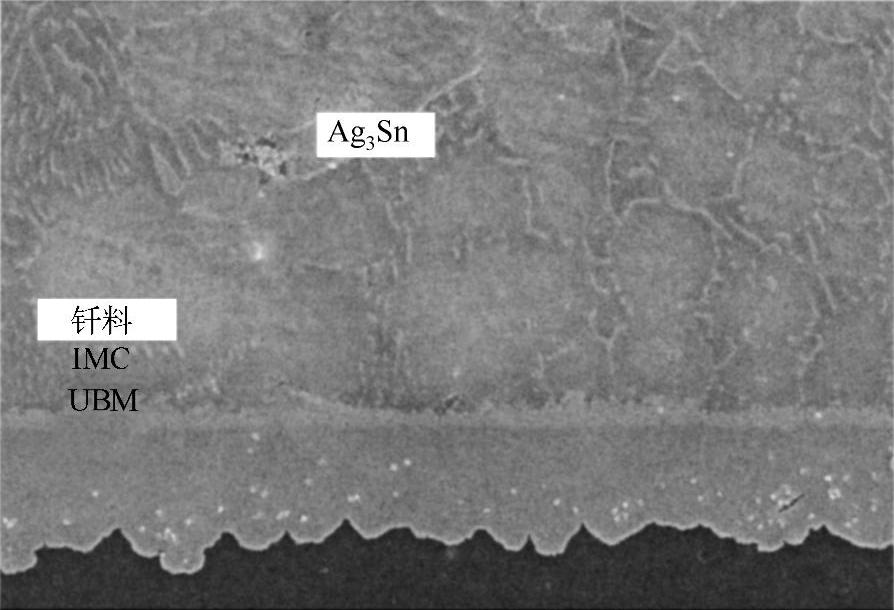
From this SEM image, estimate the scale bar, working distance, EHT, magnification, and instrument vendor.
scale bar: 50000 nm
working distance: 12.9 mm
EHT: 15 kV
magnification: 1 K X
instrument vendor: Hitachi